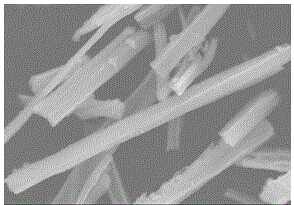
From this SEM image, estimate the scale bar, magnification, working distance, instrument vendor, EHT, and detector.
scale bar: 20000 nm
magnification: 2.5 K X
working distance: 10.5 mm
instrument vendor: Hitachi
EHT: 30 kV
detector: SE